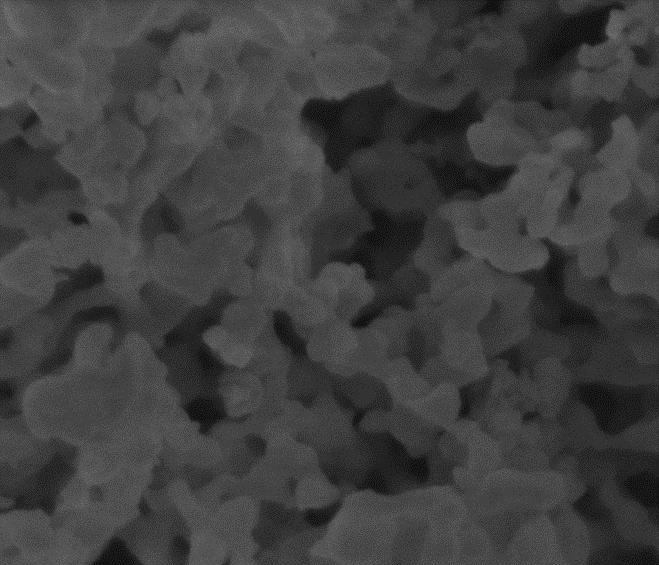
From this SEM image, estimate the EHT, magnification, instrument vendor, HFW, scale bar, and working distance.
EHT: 5 kV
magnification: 80 K X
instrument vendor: FEI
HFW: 3.73 µm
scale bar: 1000 nm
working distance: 5.8 mm